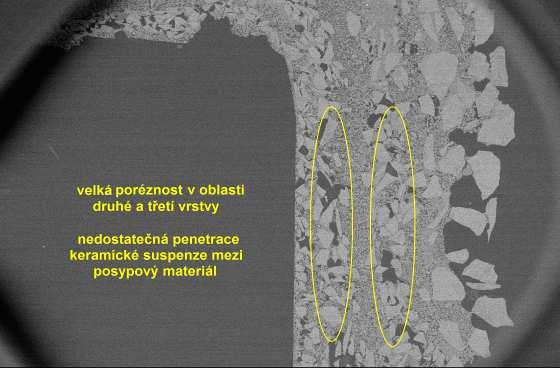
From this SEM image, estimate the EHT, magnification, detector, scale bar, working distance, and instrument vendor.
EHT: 10 kV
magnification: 0.01 K X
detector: BEC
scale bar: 2000000 nm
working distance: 45 mm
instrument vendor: JEOL